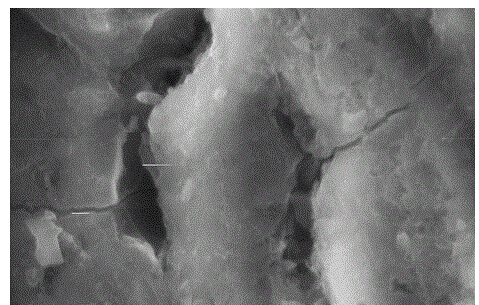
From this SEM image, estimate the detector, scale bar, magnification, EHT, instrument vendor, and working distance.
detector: SE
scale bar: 5000 nm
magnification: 10 K X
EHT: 20 kV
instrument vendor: FEI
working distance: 5.8 mm